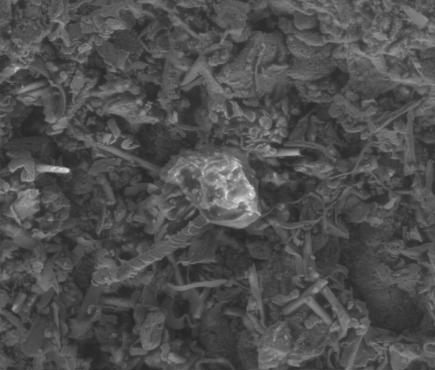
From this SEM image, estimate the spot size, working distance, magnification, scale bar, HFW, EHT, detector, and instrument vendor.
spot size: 6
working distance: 11.1 mm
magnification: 0.5 K X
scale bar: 100000 nm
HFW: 540 µm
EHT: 25 kV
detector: LFD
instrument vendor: FEI